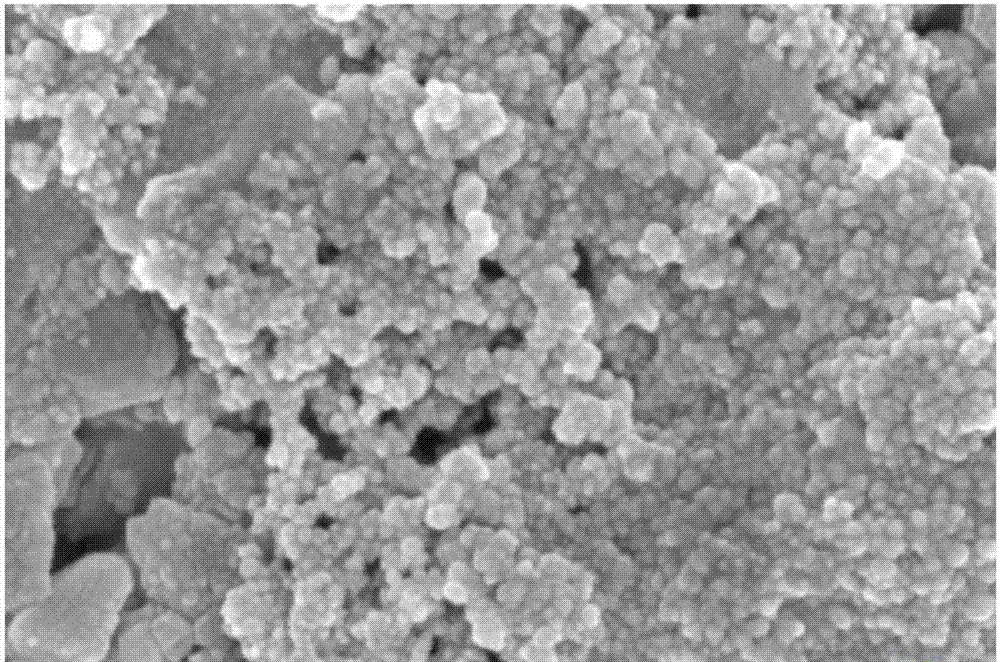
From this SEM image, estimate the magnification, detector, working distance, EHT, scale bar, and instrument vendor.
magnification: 50 K X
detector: InLens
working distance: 5.4 mm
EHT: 5 kV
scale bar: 100 nm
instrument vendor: Zeiss